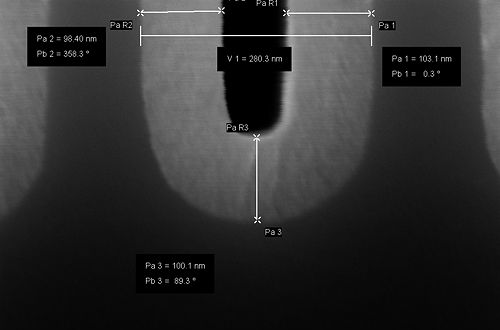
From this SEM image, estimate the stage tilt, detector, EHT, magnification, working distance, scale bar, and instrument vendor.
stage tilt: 0°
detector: InLens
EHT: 5 kV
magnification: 496.1 K X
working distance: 3.4 mm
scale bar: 20 nm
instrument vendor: Zeiss